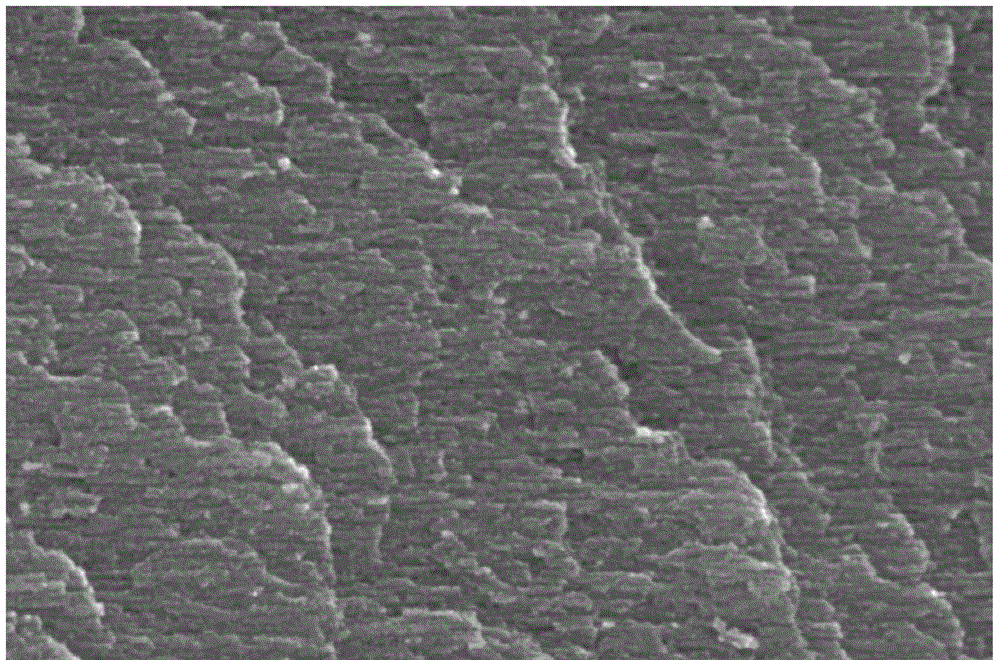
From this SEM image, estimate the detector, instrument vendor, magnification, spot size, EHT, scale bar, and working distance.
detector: TLD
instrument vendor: FEI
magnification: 240 K X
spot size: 3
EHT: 5 kV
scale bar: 500 nm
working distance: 5 mm